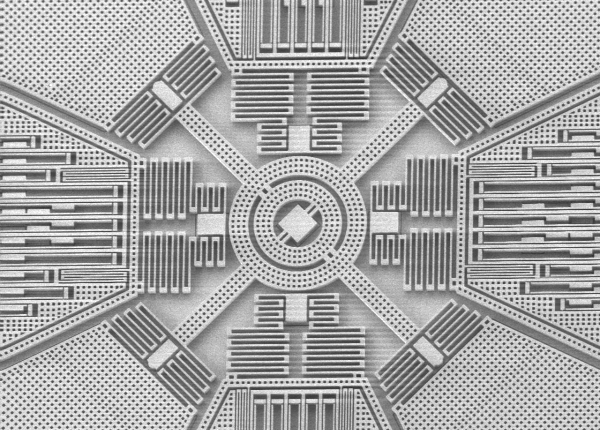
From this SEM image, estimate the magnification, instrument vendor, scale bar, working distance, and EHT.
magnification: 0.135 K X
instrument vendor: other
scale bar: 200000 nm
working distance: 4 mm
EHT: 3 kV